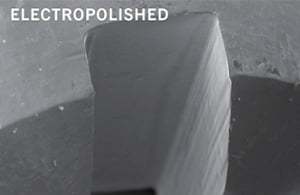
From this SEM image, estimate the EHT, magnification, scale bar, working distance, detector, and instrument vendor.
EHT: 20 kV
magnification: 0.13 K X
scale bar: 100000 nm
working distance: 13 mm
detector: SE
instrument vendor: Zeiss